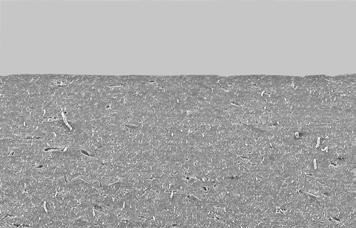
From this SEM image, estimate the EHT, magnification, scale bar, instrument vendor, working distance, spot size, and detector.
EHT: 16 kV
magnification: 0.1 K X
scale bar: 200000 nm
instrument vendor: FEI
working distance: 27.3 mm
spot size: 5.5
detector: SE